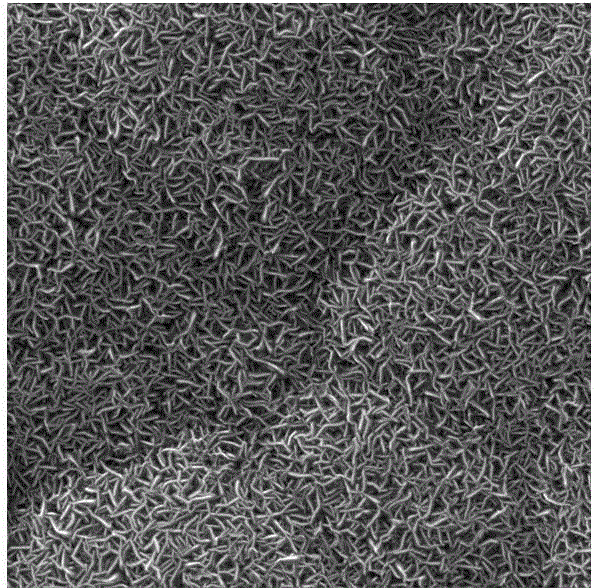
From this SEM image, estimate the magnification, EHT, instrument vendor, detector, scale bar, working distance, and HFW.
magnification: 10 K X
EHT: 30 kV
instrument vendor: Tescan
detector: SE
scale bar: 5000 nm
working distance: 8.09 mm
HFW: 20.8 µm